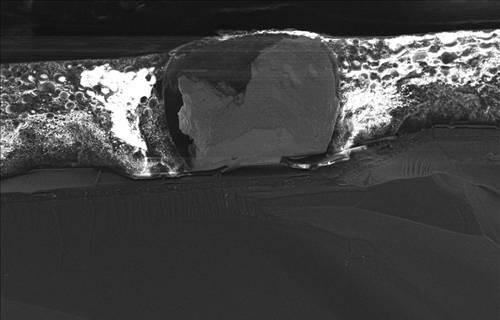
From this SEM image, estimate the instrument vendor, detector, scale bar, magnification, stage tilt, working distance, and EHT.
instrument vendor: Zeiss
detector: InLens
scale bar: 100000 nm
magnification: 0.678 K X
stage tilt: -15°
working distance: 4 mm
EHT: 15 kV